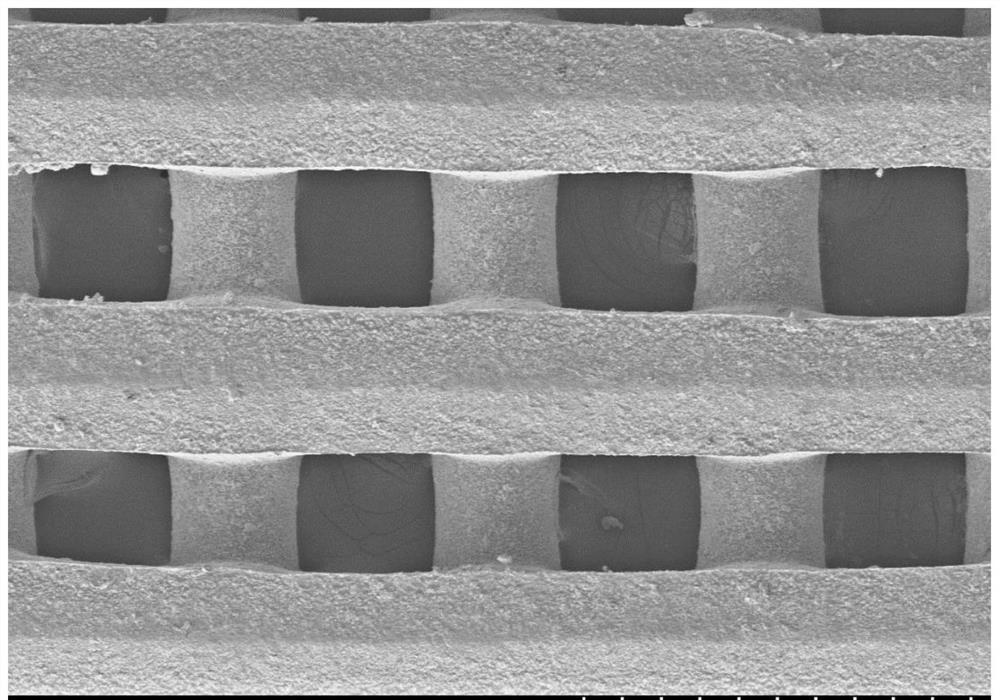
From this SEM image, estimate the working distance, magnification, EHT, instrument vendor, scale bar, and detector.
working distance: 8.1 mm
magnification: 0.1 K X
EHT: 5 kV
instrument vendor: Hitachi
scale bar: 500000 nm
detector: SE(M)